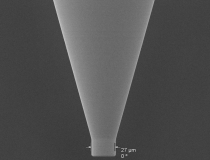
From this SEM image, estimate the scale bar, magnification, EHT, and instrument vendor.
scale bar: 50000 nm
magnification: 0.5 K X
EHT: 10 kV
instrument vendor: JEOL